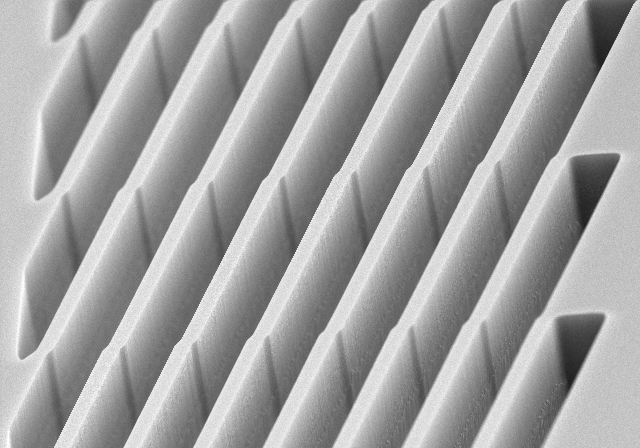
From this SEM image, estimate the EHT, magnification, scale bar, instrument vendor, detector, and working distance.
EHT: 5 kV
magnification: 15 K X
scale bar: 3000 nm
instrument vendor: Hitachi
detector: SE(M)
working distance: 2.6 mm